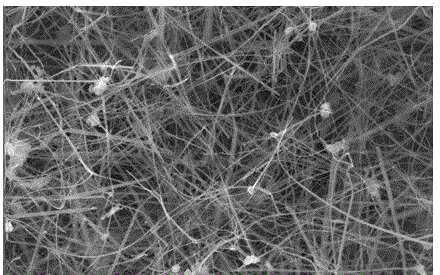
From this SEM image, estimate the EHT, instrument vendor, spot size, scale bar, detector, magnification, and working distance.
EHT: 20 kV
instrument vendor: FEI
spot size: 4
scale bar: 2000 nm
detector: SE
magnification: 10 K X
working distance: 6.1 mm